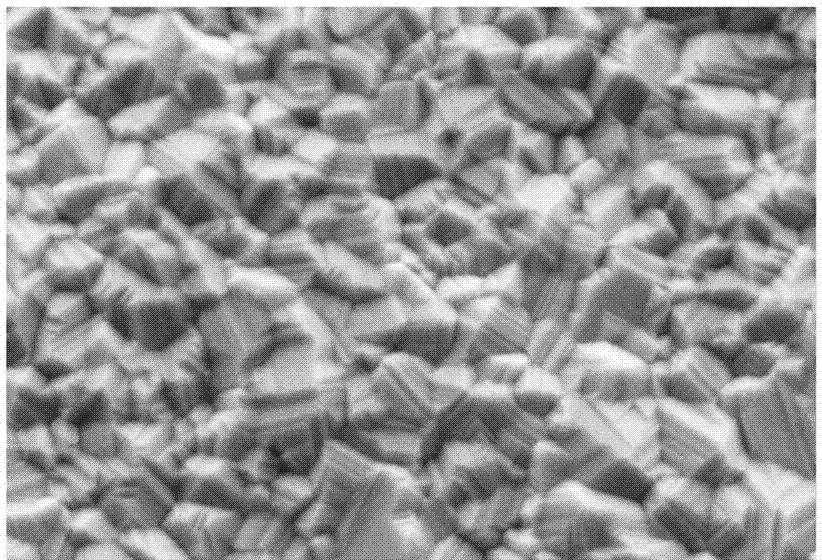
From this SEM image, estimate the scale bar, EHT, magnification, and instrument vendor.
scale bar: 10000 nm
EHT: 20 kV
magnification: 3 K X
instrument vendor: other